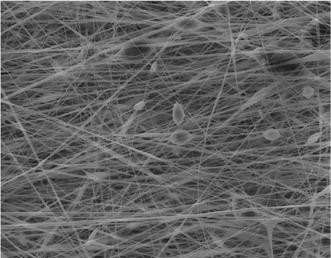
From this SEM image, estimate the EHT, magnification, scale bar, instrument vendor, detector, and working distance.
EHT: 10 kV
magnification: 5 K X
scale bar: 2000 nm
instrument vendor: Zeiss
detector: SE1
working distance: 10 mm